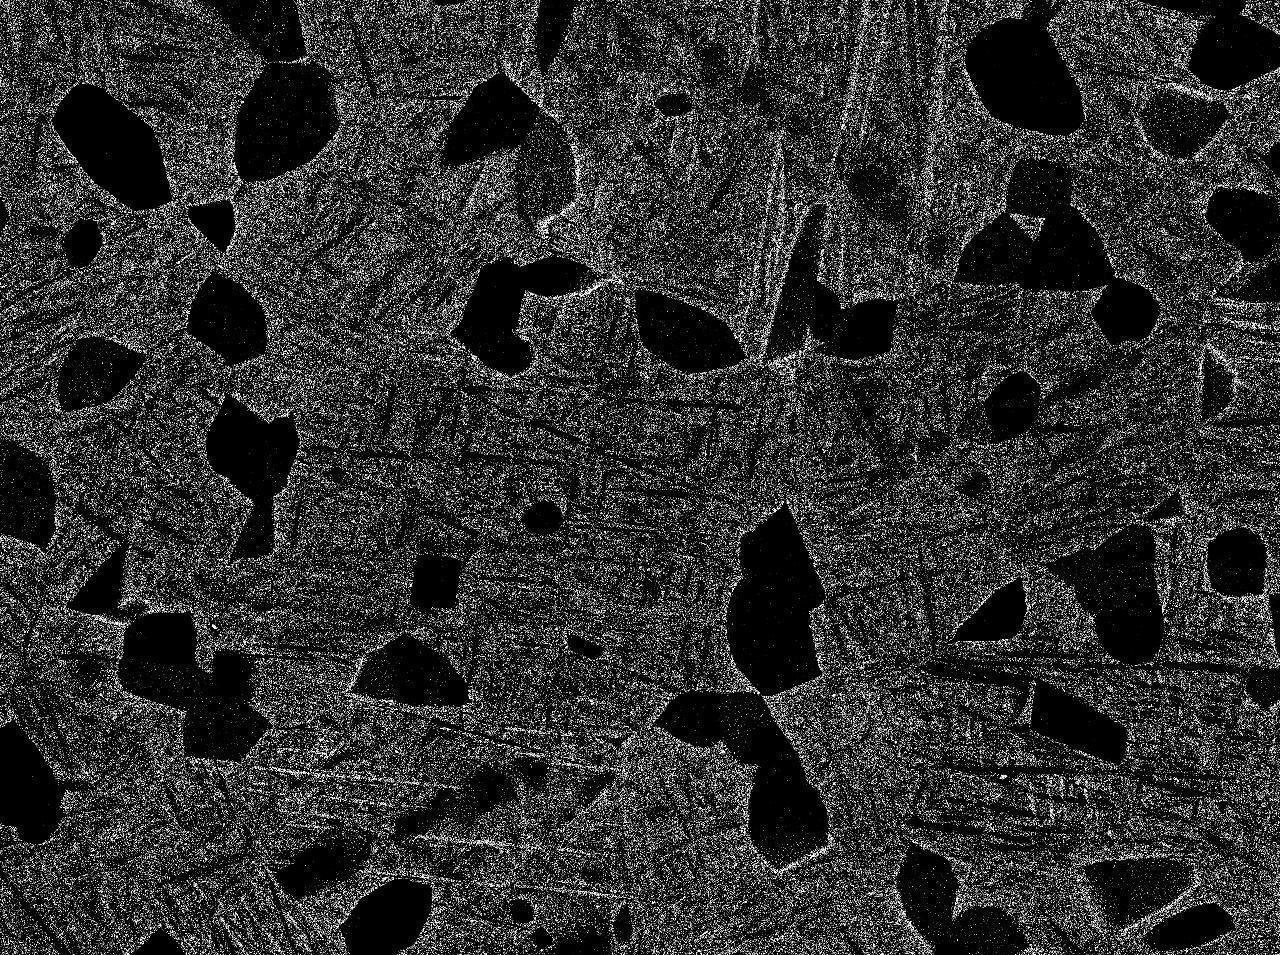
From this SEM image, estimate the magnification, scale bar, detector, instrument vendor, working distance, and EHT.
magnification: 1.5 K X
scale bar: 10000 nm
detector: COMPO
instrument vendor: JEOL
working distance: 10 mm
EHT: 15 kV